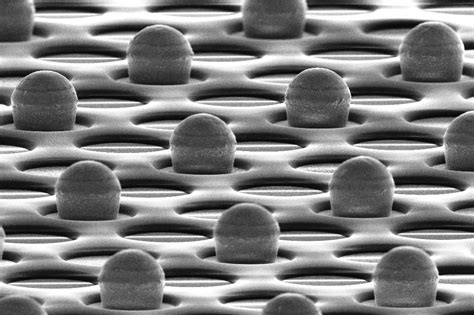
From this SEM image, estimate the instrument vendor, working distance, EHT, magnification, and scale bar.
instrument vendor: Zeiss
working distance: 9.3 mm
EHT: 10 kV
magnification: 2 K X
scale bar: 20000 nm